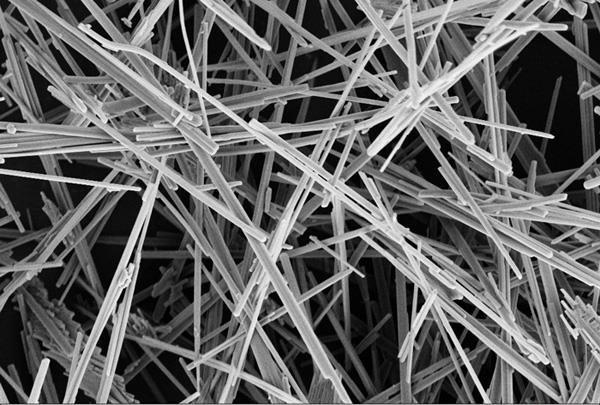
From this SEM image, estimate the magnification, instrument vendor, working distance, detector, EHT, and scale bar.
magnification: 5 K X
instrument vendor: Zeiss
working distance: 9.9 mm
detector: SE2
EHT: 4 kV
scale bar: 3000 nm